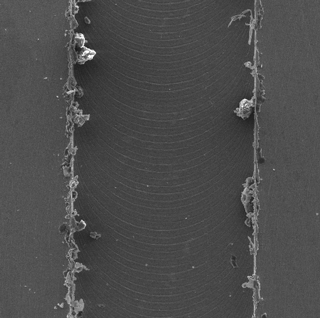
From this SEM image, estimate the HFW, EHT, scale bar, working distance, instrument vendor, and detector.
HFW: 864 µm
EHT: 20 kV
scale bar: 200000 nm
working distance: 15.77 mm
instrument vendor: Tescan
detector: SE